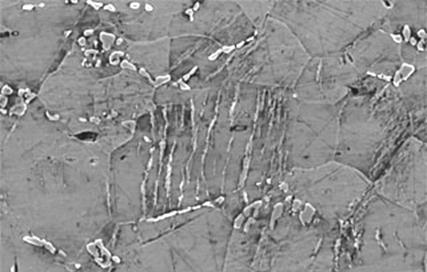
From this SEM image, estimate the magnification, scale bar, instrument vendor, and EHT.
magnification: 1 K X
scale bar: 10000 nm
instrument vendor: JEOL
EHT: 20 kV